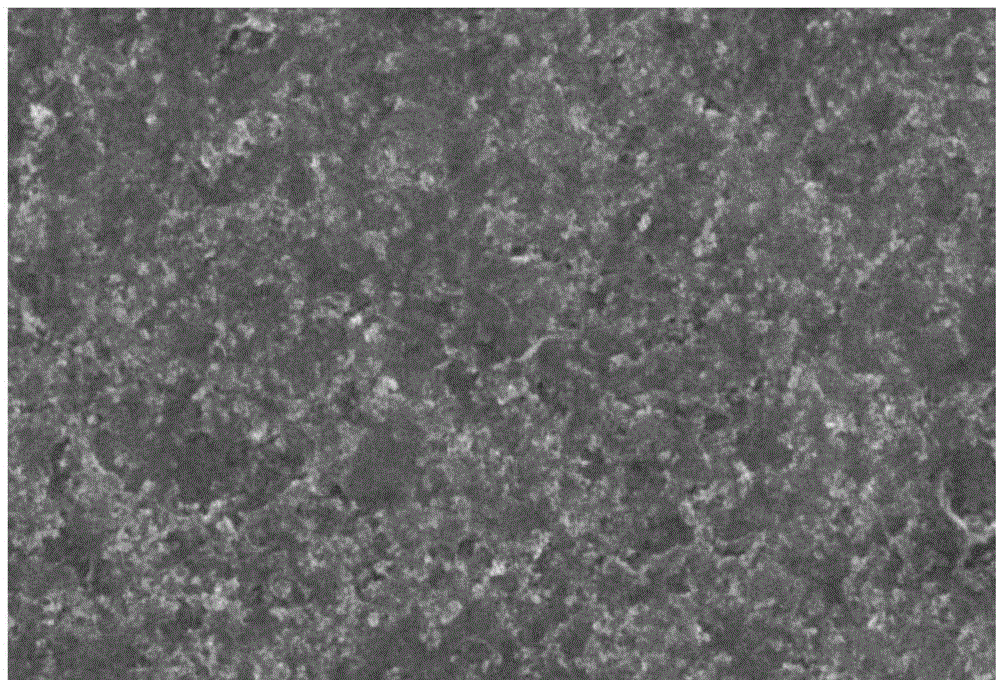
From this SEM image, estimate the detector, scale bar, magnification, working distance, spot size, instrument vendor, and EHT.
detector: SEI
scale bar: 5000 nm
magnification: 5 K X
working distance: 14 mm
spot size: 20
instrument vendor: JEOL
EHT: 25 kV